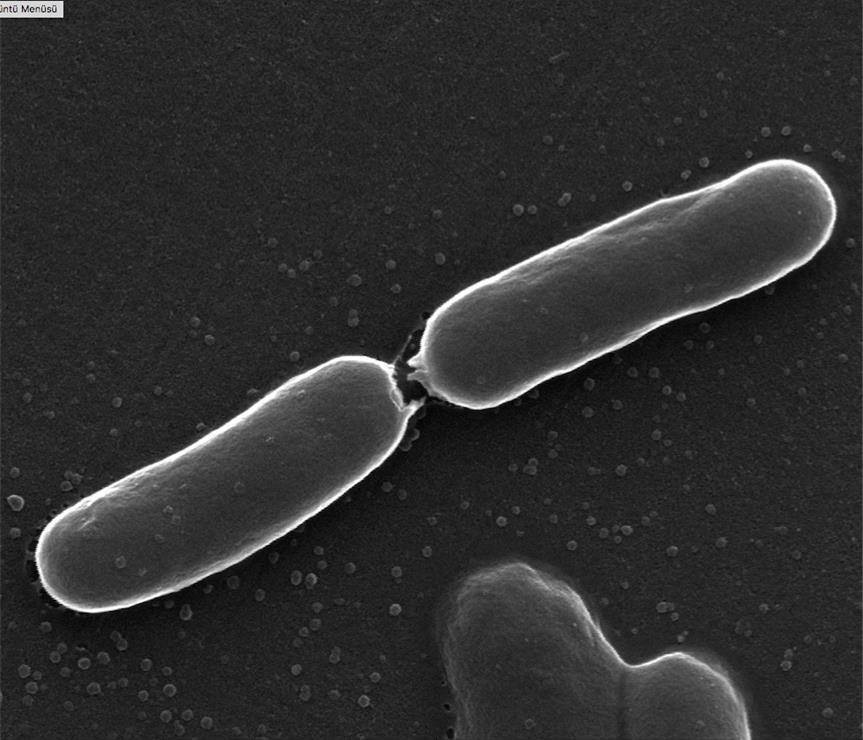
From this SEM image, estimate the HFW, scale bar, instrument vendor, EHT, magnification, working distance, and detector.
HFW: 3.66 µm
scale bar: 1000 nm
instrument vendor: FEI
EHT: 30 kV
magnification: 70 K X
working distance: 11.6 mm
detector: SE